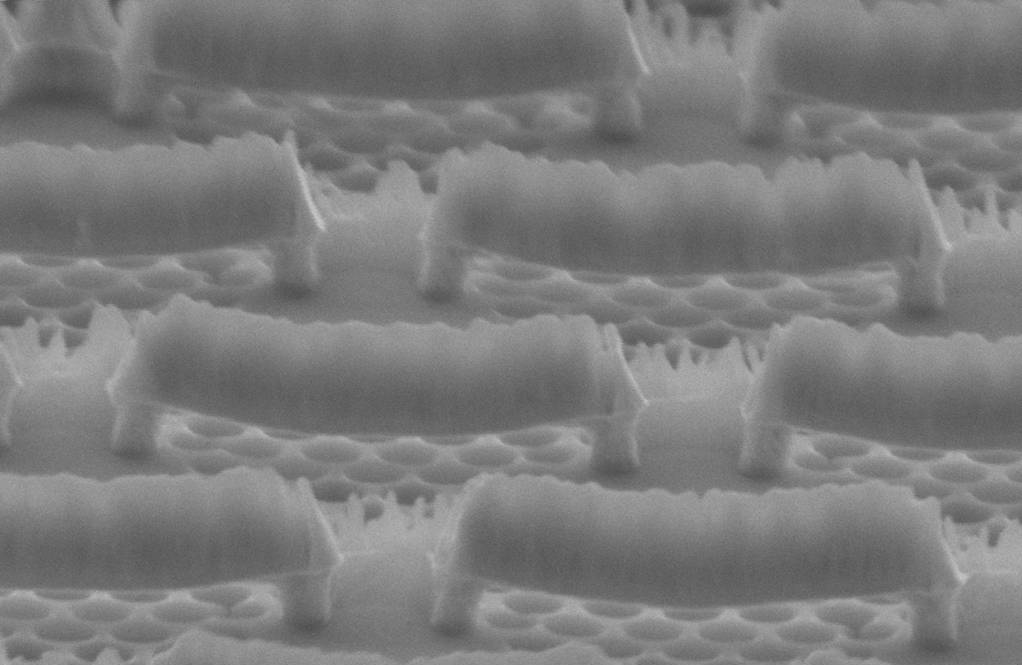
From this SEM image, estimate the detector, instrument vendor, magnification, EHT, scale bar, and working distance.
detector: InLens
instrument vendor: Zeiss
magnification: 25.74 K X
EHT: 10 kV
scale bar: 300 nm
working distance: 18 mm